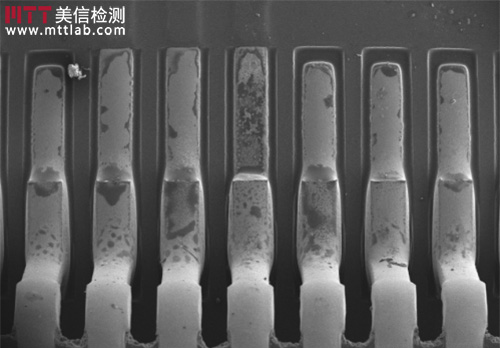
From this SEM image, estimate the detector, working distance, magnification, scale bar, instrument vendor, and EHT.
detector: SE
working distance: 10.2 mm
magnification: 0.035 K X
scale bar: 1000000 nm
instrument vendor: Hitachi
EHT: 15 kV